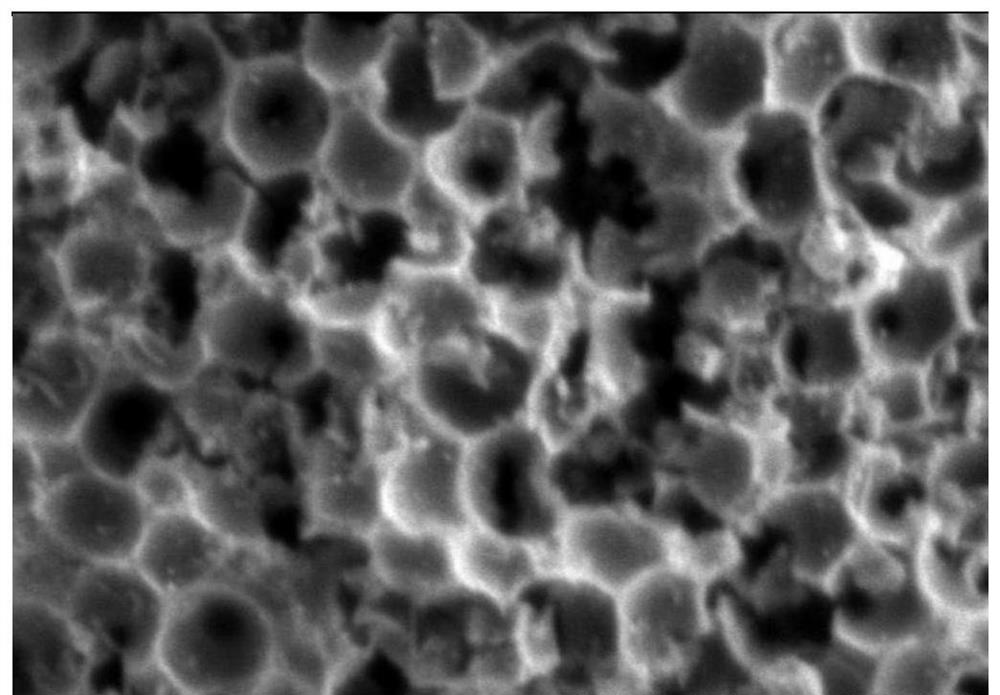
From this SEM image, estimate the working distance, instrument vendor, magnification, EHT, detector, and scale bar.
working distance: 17.8 mm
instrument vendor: Hitachi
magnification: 50 K X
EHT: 1 kV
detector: SE(L)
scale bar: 1000 nm